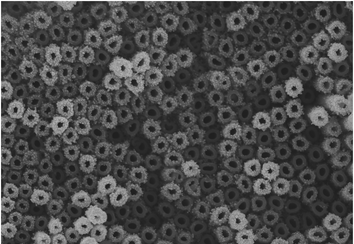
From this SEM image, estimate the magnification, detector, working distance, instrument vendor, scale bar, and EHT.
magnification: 20 K X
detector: SE(U)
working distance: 7.8 mm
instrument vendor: Hitachi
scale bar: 2000 nm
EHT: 10 kV